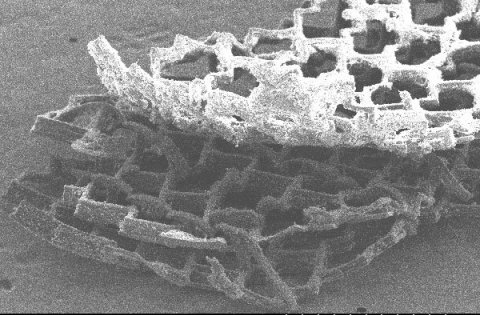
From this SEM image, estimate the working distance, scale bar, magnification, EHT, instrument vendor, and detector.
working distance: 19.4 mm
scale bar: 1000000 nm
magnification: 0.042 K X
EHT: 20 kV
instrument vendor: Hitachi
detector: SE